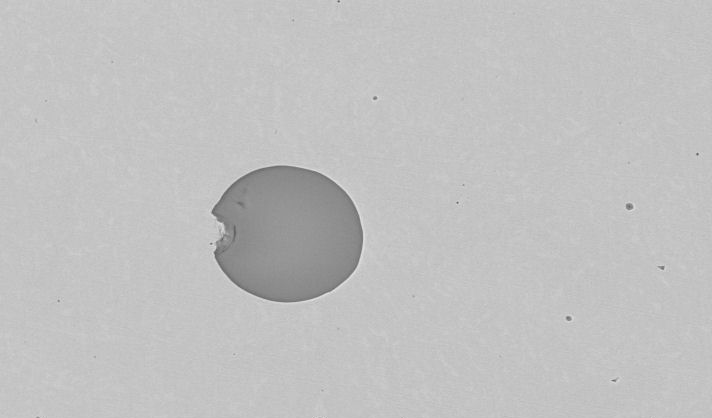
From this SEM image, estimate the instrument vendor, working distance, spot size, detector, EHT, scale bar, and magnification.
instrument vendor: FEI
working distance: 10 mm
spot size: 5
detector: BSE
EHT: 20 kV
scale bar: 20000 nm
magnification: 1.5 K X